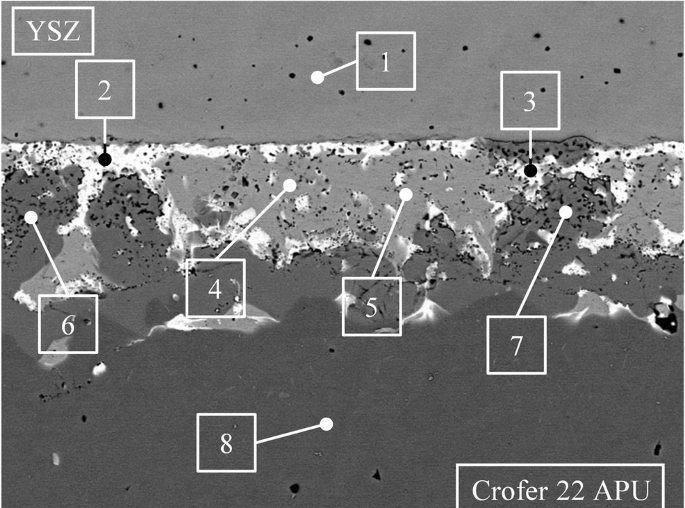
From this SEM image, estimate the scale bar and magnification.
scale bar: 10000 nm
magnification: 2 K X